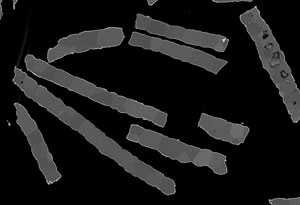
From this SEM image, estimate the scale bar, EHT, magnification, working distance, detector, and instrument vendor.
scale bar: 50000 nm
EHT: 1 kV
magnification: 1 K X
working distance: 8 mm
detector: LA100(U)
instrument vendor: Hitachi